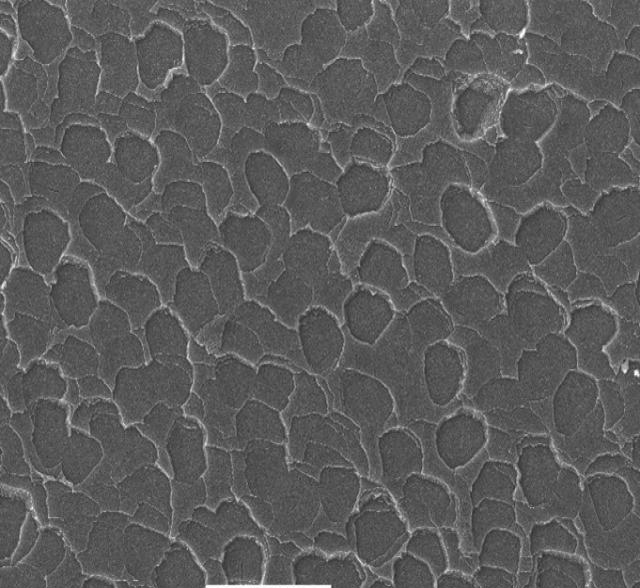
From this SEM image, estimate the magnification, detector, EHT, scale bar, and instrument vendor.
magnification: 0.25 K X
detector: SEI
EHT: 30 kV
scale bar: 100000 nm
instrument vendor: JEOL